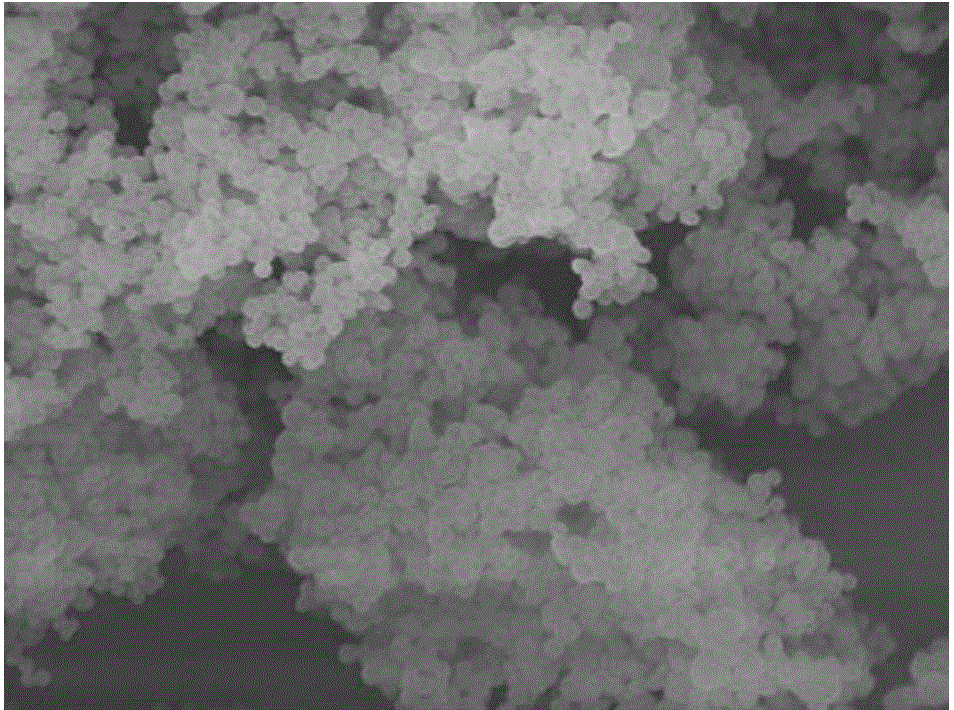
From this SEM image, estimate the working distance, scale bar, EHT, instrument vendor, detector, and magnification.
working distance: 8.2 mm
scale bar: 1000 nm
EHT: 10 kV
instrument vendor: JEOL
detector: SEI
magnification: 10 K X